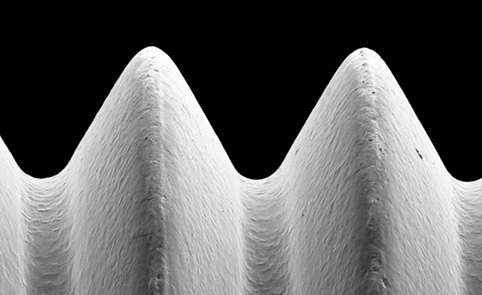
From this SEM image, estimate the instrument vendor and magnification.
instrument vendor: other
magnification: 0.3 K X